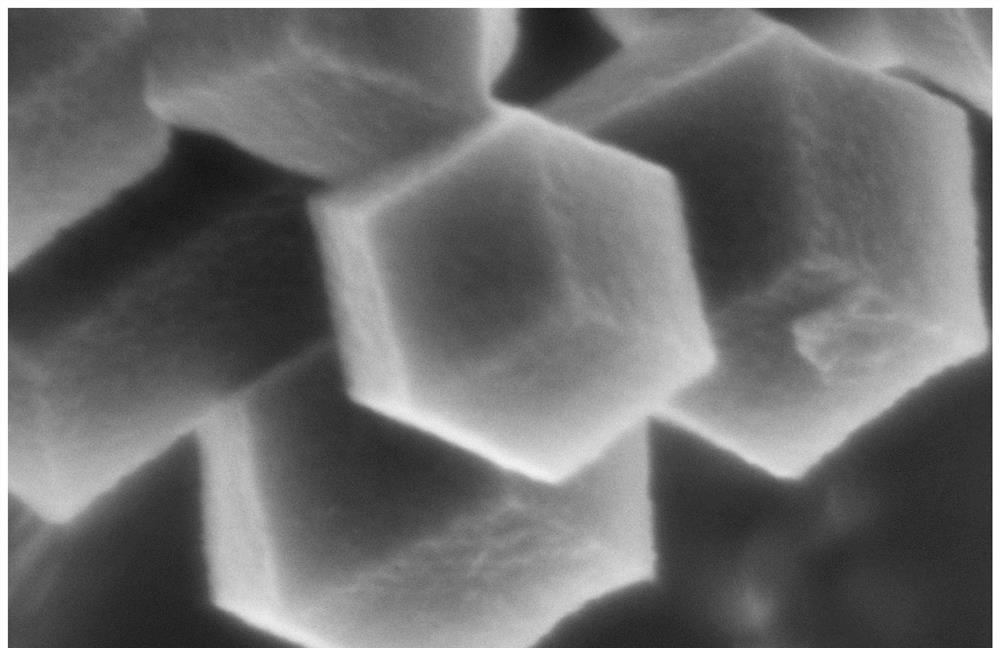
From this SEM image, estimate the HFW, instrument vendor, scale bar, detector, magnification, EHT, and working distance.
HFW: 0.635 µm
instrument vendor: Thermo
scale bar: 100 nm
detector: TLD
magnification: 200 K X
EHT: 2 kV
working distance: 2.5 mm